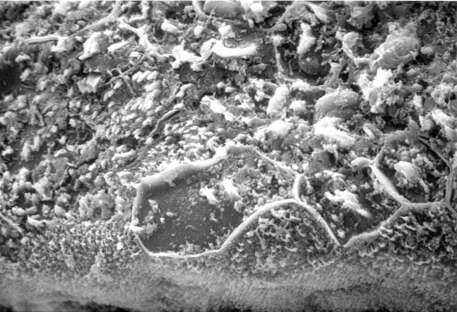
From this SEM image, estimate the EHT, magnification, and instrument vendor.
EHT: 20 kV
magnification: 1.1 K X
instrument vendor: JEOL